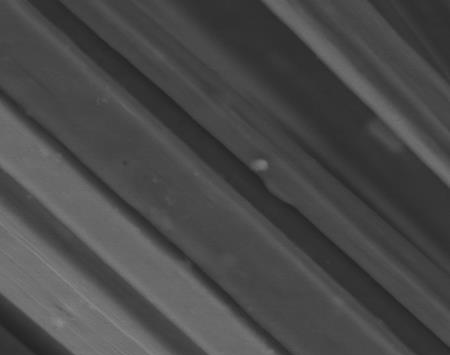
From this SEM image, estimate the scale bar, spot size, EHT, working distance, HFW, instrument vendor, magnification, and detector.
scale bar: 20000 nm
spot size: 4.5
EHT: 15 kV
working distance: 5.8 mm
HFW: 59.7 µm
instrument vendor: FEI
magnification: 5 K X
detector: LVD